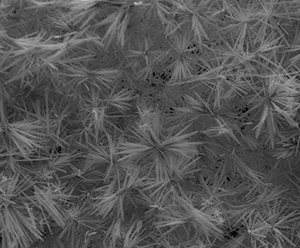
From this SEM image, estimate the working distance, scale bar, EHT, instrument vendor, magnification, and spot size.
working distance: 10.6 mm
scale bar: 30000 nm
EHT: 20 kV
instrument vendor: FEI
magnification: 2.5 K X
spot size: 3